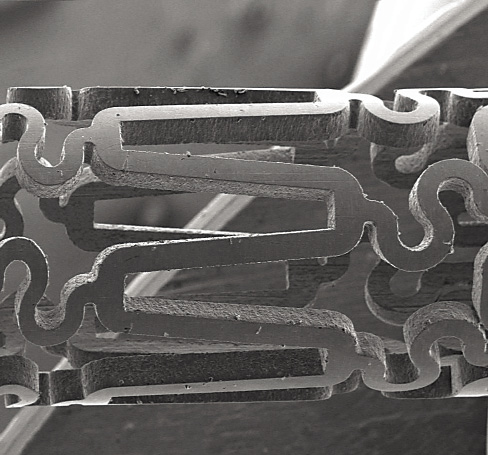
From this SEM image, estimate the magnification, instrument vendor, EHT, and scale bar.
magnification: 0.04 K X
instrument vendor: JEOL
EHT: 15 kV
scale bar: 500000 nm